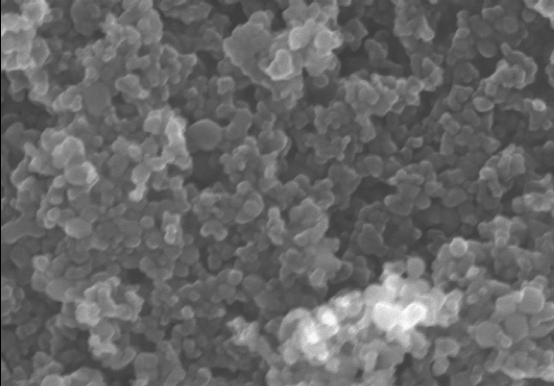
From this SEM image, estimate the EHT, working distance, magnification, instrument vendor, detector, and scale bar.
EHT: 10 kV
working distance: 8.6 mm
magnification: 120 K X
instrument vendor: Hitachi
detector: SE(U)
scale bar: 400 nm